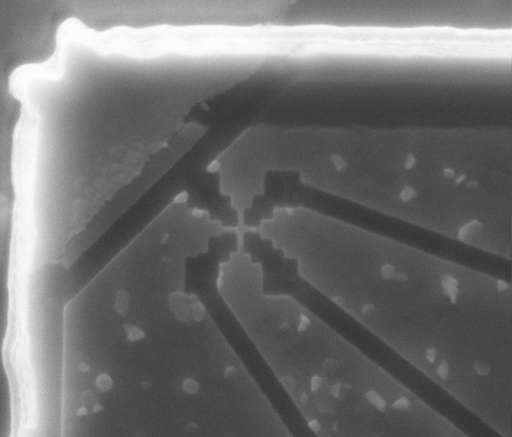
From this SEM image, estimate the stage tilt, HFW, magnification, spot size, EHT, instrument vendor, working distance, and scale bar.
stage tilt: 0°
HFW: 4.68 µm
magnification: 65 K X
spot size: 2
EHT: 10 kV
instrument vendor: FEI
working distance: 5.16 mm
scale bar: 500 nm